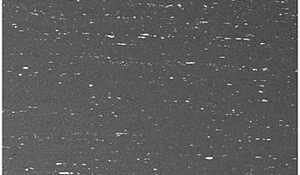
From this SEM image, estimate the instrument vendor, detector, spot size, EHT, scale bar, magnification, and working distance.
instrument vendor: FEI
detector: BSE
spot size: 4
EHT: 20 kV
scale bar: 100000 nm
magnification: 0.5 K X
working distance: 7 mm